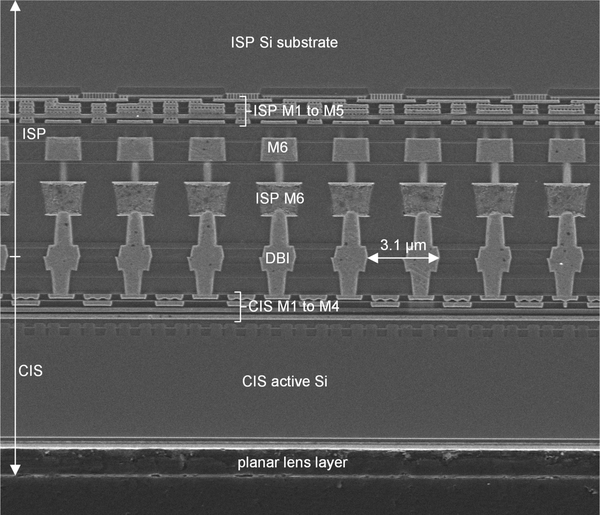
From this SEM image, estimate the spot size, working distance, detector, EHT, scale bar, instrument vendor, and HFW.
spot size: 2.5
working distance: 5.5 mm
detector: ETD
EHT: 5 kV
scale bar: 10000 nm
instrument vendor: FEI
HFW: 25.4 µm